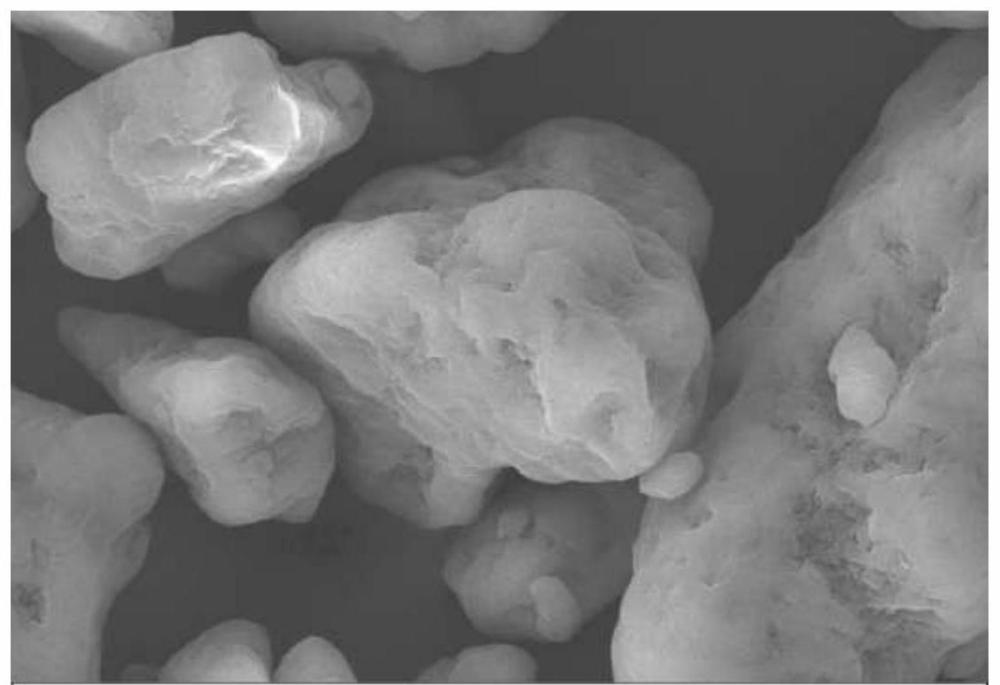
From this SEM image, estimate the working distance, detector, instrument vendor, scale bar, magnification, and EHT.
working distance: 7.9 mm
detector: SE2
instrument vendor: Zeiss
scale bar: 2000 nm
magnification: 8 K X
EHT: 15 kV